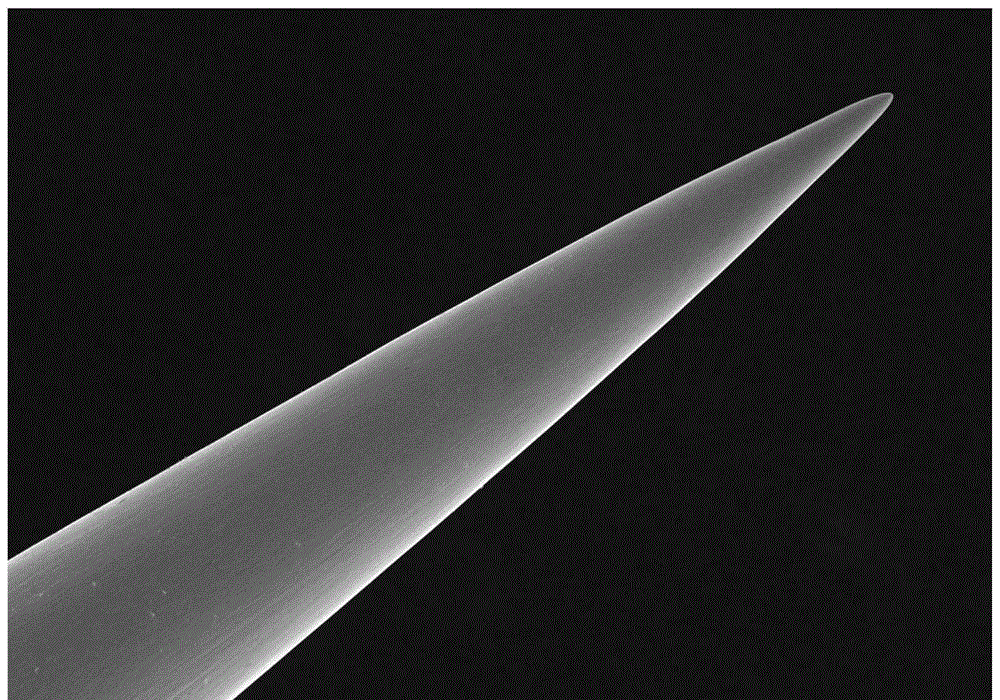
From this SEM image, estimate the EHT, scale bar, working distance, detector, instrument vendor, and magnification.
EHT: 15 kV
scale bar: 200000 nm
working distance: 12 mm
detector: SE(L)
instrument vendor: Hitachi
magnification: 0.2 K X